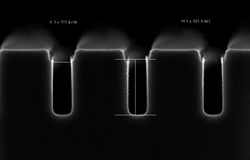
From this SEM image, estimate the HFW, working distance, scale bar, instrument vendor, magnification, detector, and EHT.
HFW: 1.501 µm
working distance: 3.2 mm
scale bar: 100 nm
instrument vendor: Zeiss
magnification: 200 K X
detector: InLens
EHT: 2 kV